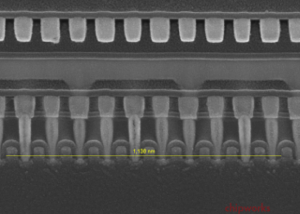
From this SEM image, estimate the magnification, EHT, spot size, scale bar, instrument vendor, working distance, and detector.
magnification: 100 K X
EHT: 10 kV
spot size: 3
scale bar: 200 nm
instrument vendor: FEI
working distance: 5.2 mm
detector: TLD